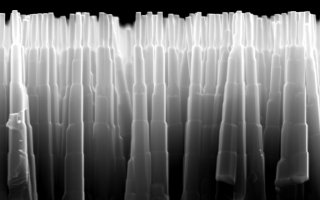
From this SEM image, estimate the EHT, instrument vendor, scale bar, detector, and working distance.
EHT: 25 kV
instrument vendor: Zeiss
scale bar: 1000 nm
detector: SE1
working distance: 6 mm